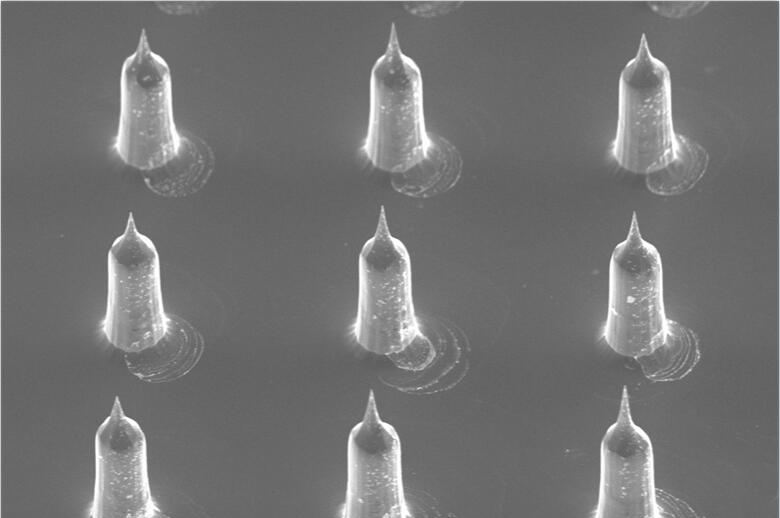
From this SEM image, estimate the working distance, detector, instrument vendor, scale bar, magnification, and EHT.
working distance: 37 mm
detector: SE1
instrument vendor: Zeiss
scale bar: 200000 nm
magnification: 0.06 K X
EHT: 10 kV